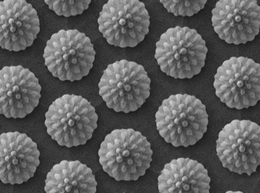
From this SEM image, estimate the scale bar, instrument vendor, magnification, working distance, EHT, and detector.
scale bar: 1000 nm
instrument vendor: JEOL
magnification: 10 K X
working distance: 8 mm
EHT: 1.5 kV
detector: LED